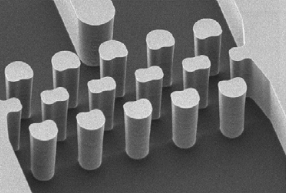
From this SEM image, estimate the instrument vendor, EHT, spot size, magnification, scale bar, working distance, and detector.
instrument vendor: FEI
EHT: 5 kV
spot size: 3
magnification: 0.55 K X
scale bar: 50000 nm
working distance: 15.4 mm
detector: SE